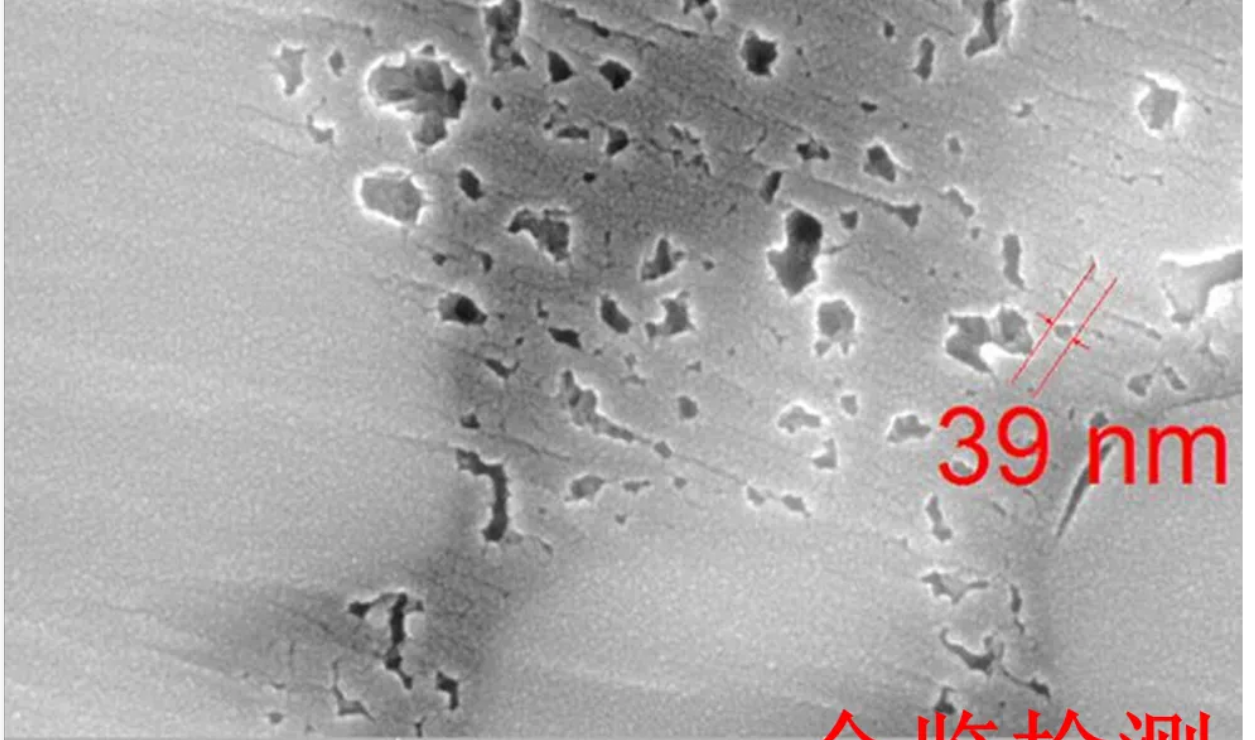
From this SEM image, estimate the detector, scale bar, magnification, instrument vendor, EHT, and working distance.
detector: InBeam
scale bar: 500 nm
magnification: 100 K X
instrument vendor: Tescan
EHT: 20 kV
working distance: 6.03 mm